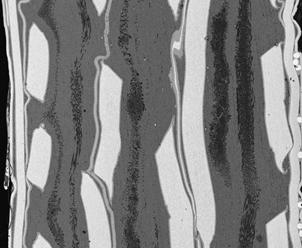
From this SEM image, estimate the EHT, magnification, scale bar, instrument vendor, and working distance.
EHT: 20 kV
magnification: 0.08 K X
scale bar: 1000000 nm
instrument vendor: FEI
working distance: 10.5 mm